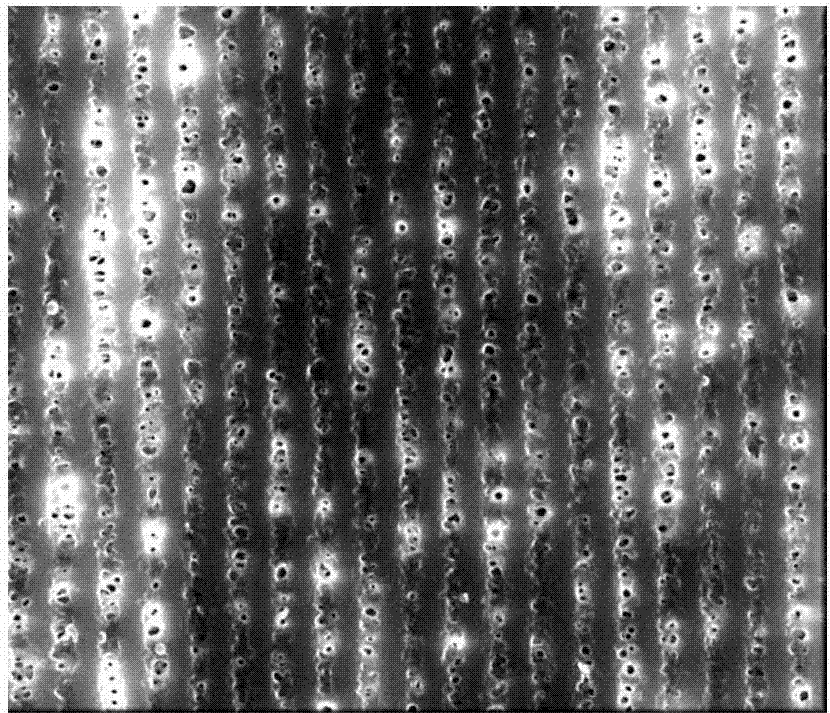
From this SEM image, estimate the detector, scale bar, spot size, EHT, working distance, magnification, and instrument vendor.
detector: TLD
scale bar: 5000 nm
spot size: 3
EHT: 10 kV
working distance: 5.3 mm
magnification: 20 K X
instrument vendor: FEI